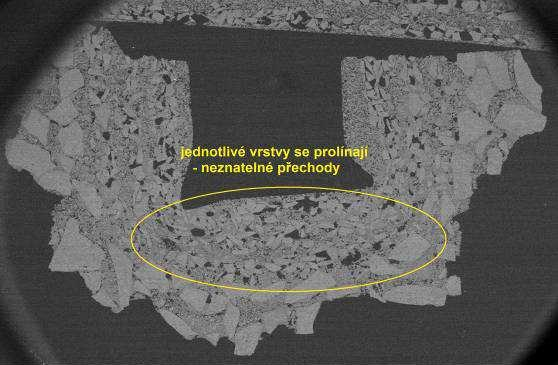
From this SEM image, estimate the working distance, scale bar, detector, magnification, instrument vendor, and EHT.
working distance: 45 mm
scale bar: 2000000 nm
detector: BEC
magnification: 0.01 K X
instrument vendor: JEOL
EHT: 10 kV